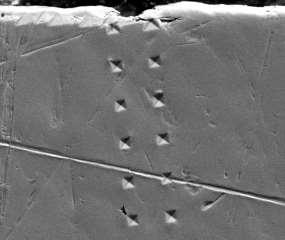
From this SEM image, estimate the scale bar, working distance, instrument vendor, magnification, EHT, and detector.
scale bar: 50000 nm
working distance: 10 mm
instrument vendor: FEI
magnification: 1 K X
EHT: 20 kV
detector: ETD